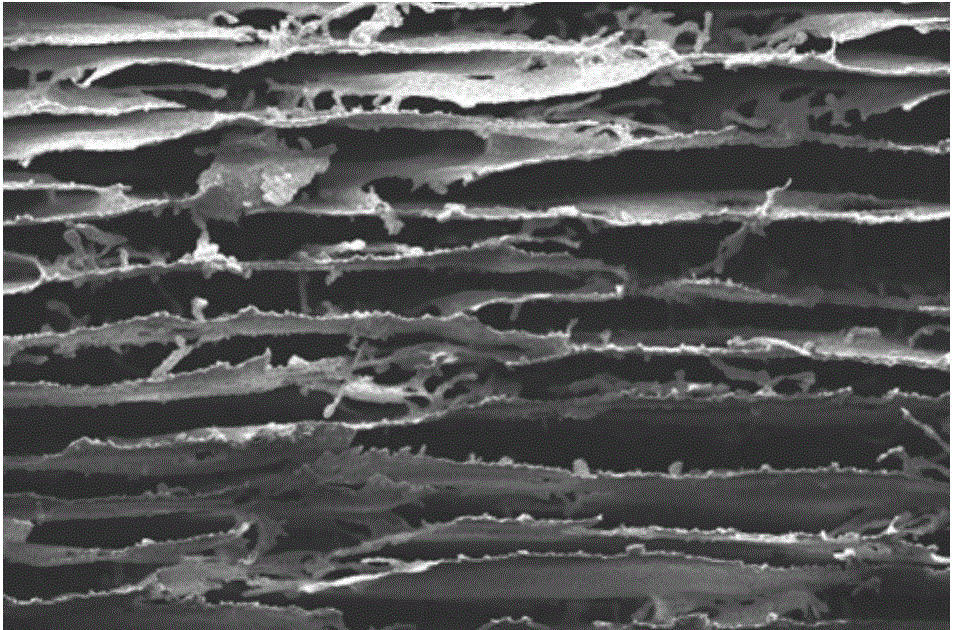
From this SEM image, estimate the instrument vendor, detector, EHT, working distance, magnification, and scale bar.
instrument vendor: Zeiss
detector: InLens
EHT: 7 kV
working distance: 6.8 mm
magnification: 0.488 K X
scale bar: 30000 nm